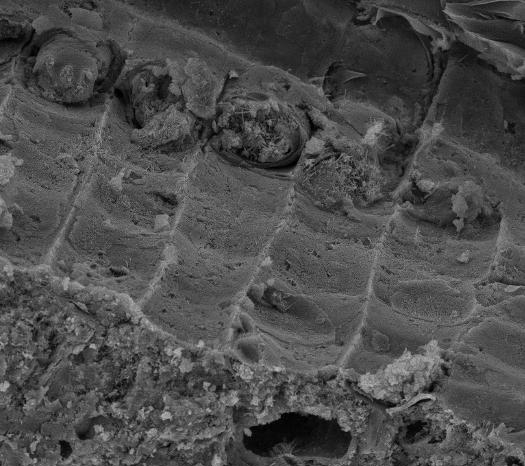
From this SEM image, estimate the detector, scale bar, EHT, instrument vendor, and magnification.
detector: SE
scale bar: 20000 nm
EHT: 15 kV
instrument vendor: Tescan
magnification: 2 K X